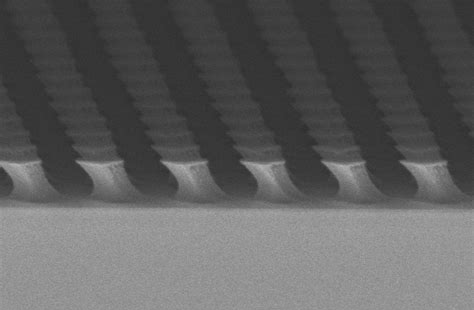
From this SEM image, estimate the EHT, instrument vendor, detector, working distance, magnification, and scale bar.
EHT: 5 kV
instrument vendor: Zeiss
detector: InLens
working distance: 8 mm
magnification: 100 K X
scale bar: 200 nm